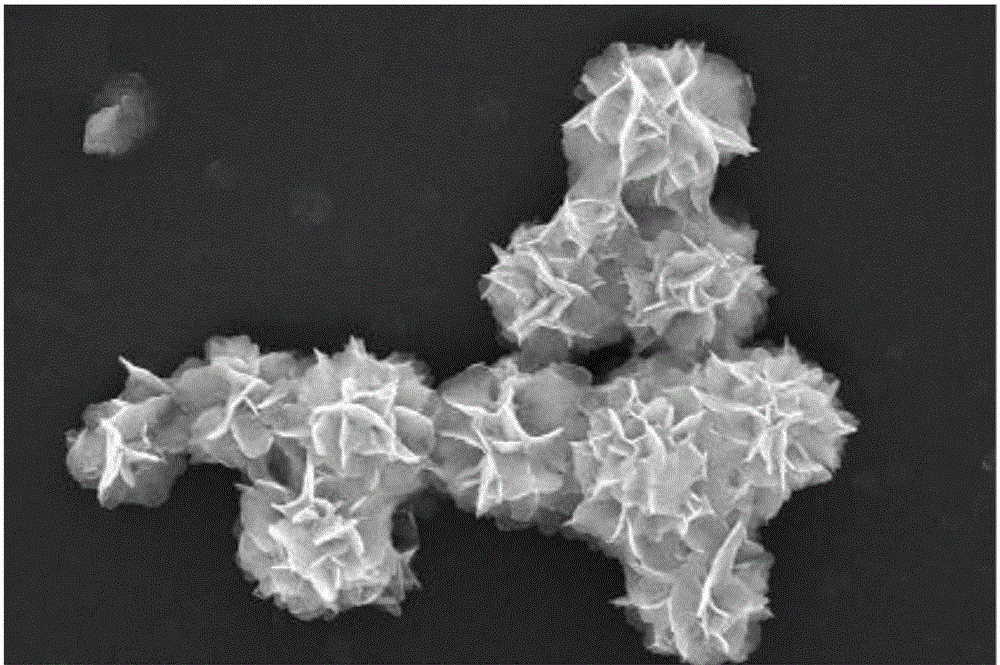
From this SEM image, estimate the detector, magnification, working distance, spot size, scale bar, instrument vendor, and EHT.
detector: ETD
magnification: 42.977 K X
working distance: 14.9 mm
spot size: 2.5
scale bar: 4000 nm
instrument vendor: FEI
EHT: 20 kV